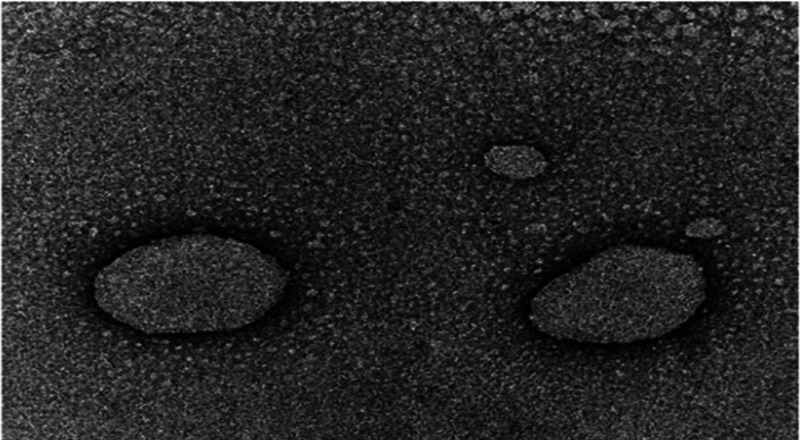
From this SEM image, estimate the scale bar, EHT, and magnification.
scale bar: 200 nm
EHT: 200 kV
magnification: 15 K X